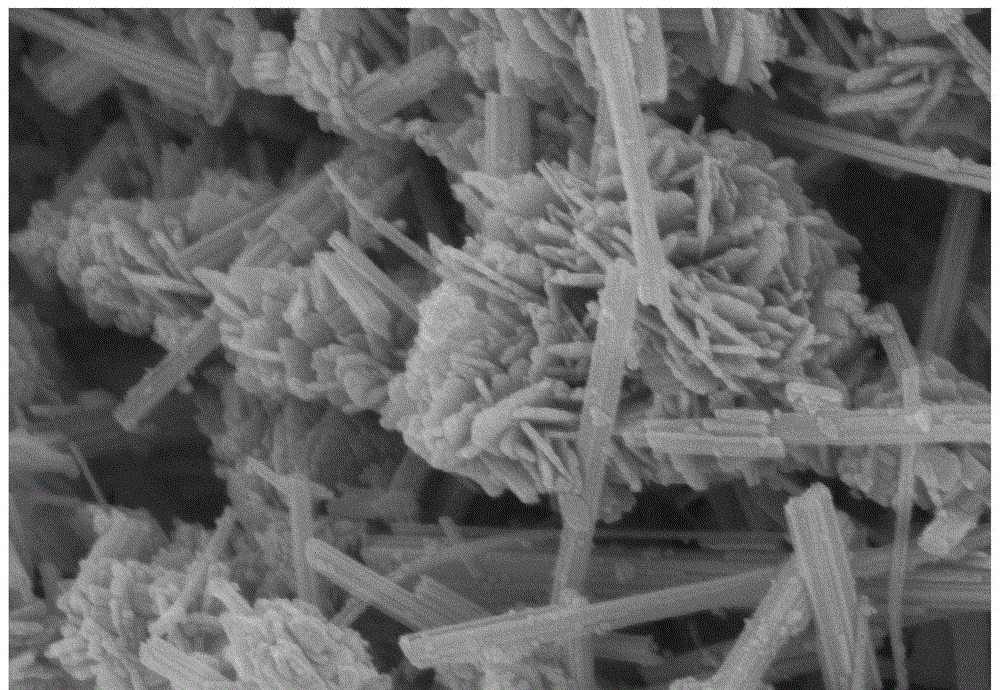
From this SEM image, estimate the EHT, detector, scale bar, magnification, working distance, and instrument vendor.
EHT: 5 kV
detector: SE(U)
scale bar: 500 nm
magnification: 60 K X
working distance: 8.4 mm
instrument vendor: Hitachi